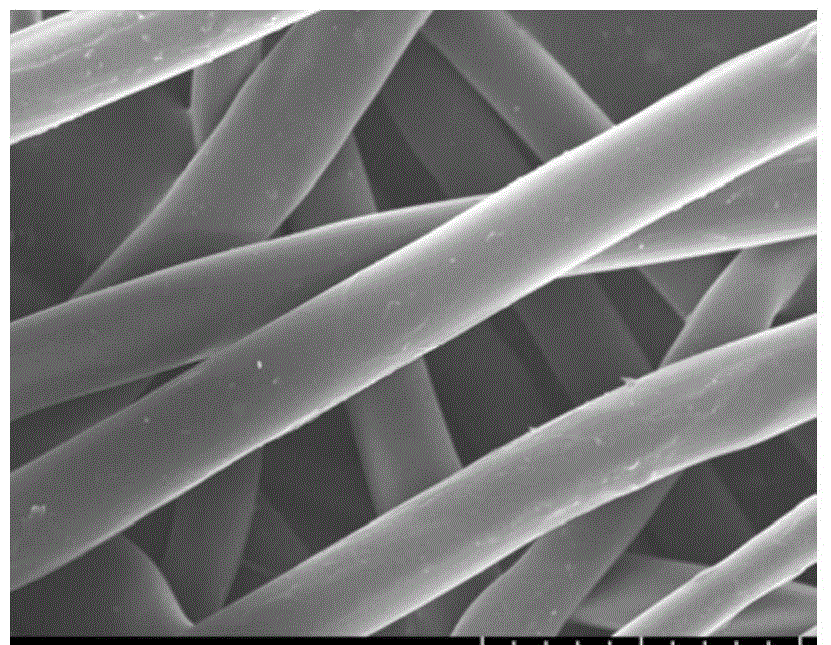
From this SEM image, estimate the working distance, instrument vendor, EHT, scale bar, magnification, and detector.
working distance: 8.3 mm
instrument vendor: Hitachi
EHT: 15 kV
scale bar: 50000 nm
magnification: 1 K X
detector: SE(UL)